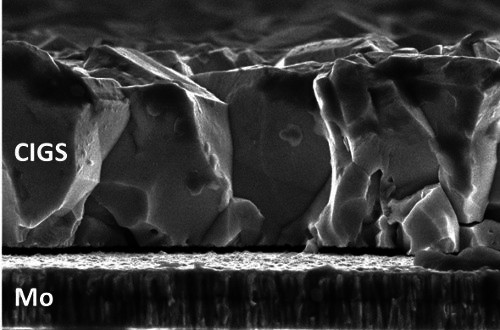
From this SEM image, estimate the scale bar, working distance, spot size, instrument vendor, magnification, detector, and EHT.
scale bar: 1000 nm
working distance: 5 mm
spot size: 3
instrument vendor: FEI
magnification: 50 K X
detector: TLD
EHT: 5 kV